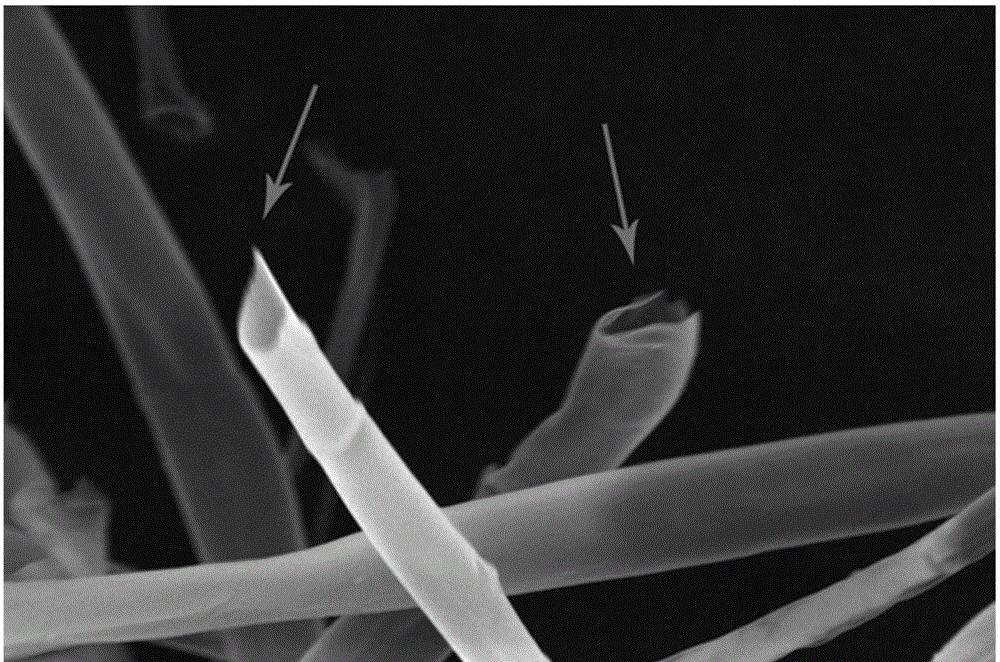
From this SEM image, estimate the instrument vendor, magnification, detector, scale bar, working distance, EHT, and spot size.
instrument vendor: FEI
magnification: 5 K X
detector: ETD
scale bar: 30000 nm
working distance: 7.8 mm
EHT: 20 kV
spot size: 4